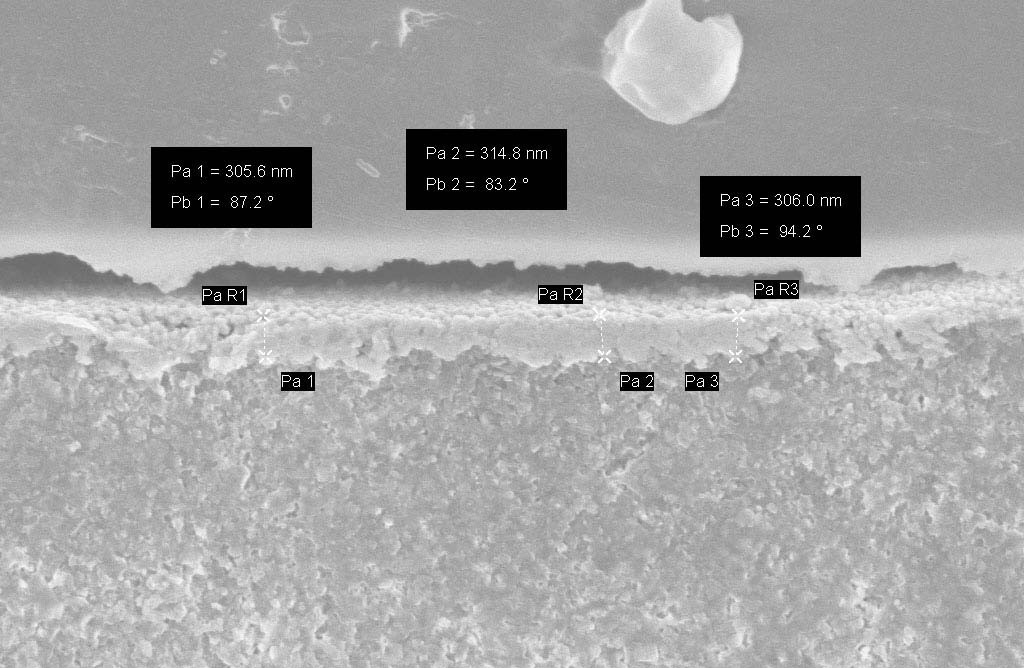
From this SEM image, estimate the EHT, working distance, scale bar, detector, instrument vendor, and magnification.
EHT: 5 kV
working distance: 5 mm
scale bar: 1000 nm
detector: InLens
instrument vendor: Zeiss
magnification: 50 K X